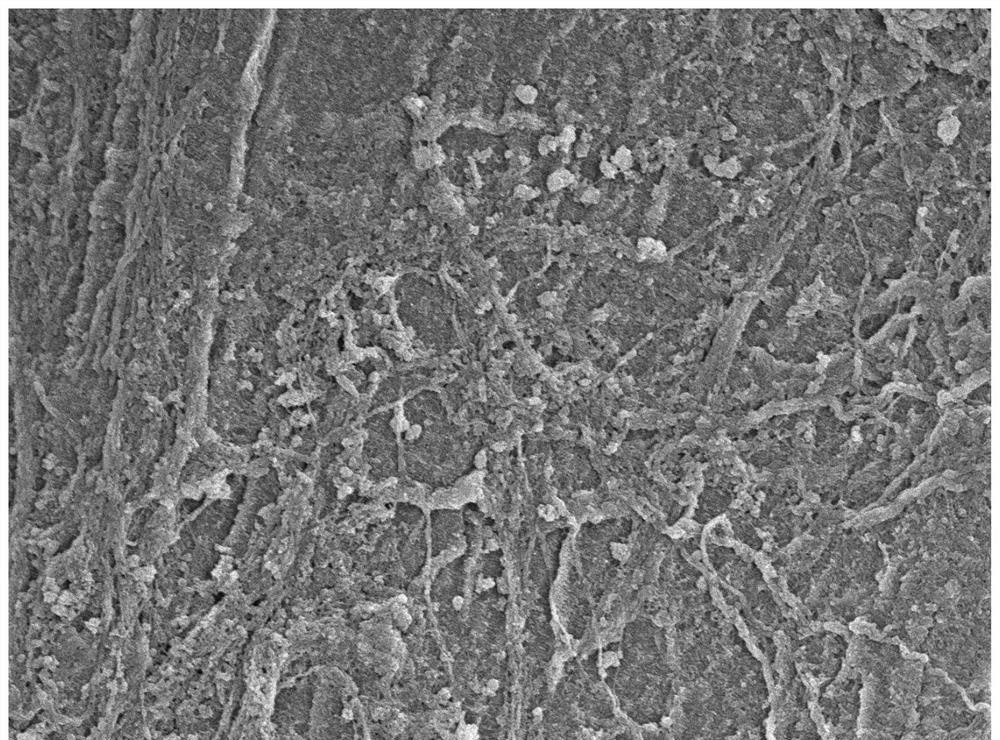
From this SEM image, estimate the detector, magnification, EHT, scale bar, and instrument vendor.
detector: SEI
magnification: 5 K X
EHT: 5 kV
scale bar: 1000 nm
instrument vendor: JEOL